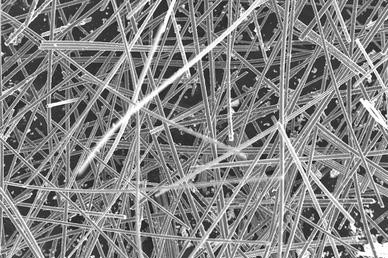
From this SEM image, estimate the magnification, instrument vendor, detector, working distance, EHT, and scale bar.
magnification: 0.2 K X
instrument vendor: Zeiss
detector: SE1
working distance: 21 mm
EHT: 20 kV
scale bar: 100000 nm